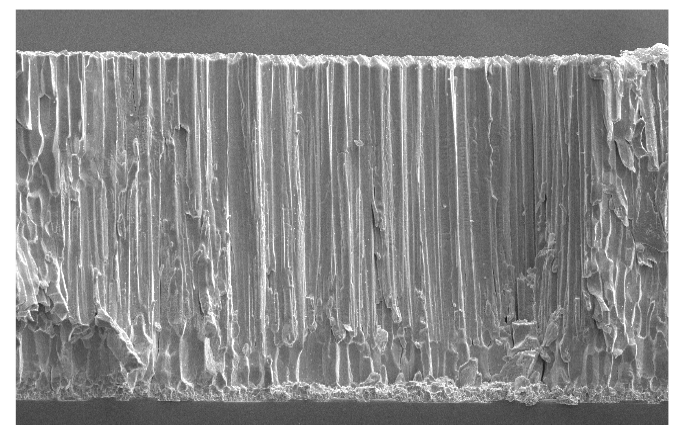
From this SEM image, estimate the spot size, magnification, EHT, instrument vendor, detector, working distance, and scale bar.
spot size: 2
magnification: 0.5 K X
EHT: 10 kV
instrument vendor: FEI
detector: SE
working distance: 16.5 mm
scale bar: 50000 nm